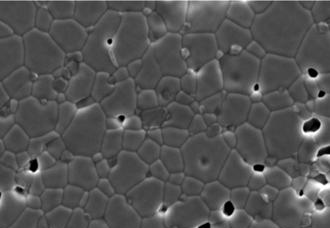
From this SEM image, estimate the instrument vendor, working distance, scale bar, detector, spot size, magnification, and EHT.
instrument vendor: FEI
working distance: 10.7 mm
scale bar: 5000 nm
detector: SE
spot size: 4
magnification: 5 K X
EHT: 15 kV